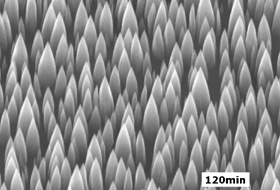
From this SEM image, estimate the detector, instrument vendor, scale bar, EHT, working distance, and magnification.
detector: SEI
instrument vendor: JEOL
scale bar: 100 nm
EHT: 20 kV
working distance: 7.1 mm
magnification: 50 K X